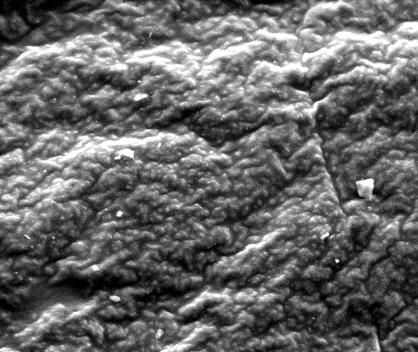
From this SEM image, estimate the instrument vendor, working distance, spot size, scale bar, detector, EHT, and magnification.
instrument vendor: FEI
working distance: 11.8 mm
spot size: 5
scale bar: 100000 nm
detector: ETD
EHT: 30 kV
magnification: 0.5 K X